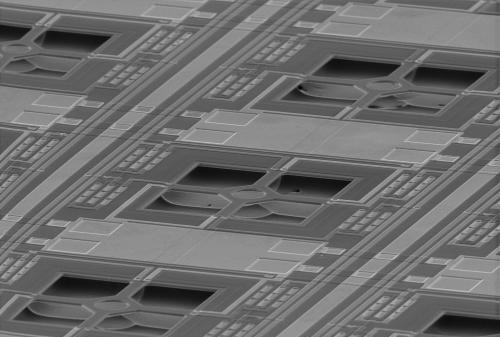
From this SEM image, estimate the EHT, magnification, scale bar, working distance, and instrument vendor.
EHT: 25 kV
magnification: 0.1 K X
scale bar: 200000 nm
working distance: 48 mm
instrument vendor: Tescan